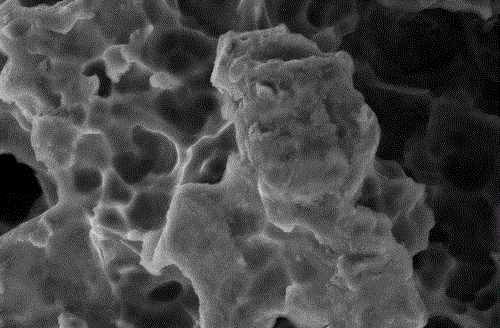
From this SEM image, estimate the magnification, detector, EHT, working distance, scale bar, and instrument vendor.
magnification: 20 K X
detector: SE2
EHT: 5 kV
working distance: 12.1 mm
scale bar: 200 nm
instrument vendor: Zeiss